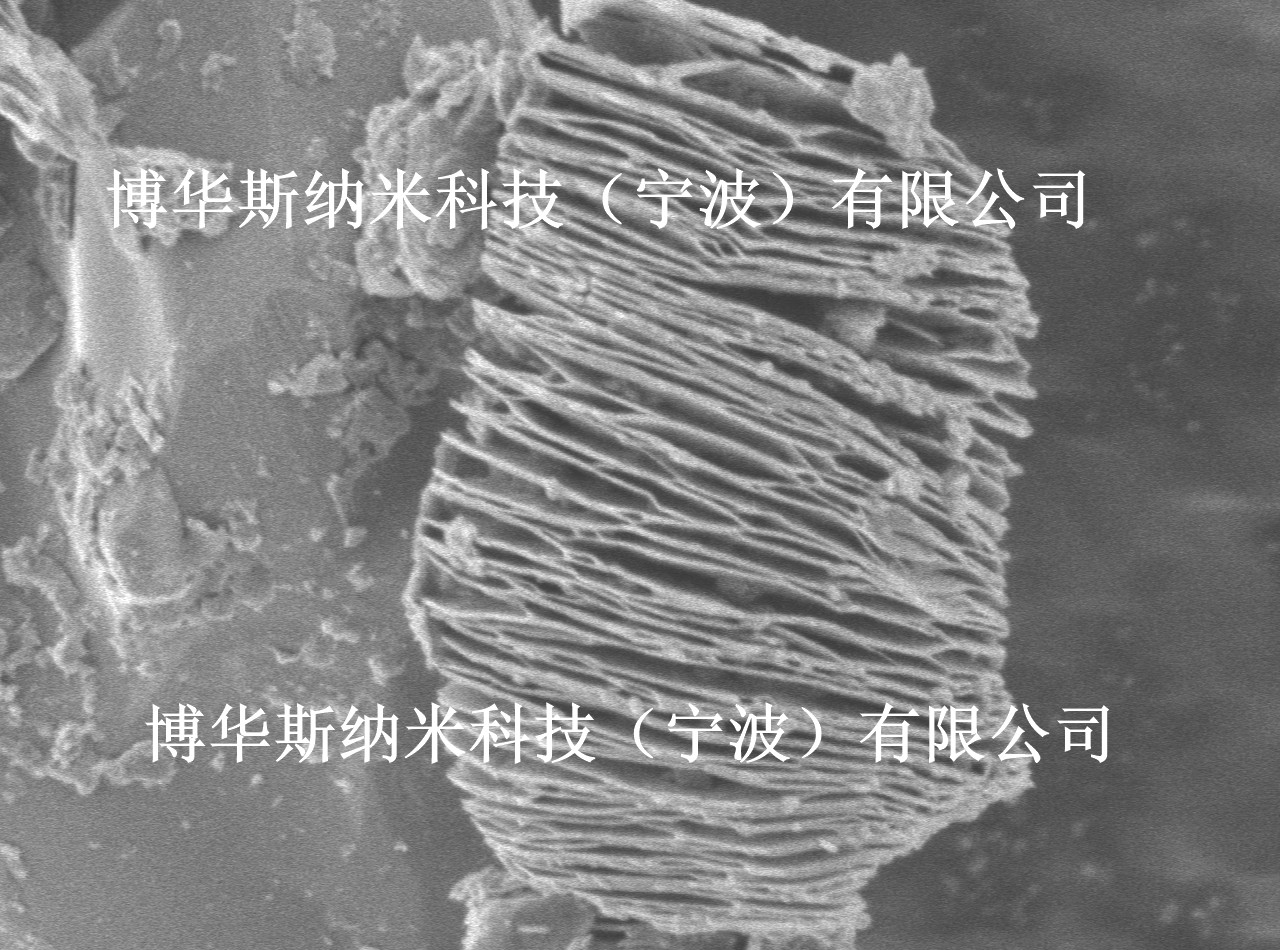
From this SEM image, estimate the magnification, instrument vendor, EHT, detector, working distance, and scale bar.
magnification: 17 K X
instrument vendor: JEOL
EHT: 2 kV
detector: LED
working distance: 10.3 mm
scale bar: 1000 nm